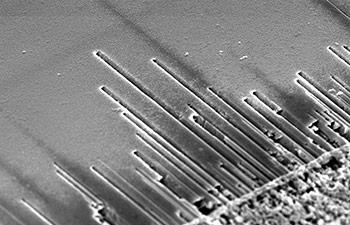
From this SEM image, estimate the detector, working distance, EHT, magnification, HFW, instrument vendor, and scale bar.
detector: TLD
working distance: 4.1 mm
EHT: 5 kV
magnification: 17.5 K X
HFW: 8.53 µm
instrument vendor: FEI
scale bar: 3000 nm